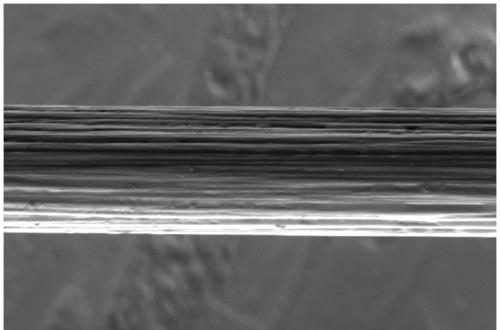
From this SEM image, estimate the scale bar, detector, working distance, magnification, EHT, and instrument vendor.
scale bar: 4000 nm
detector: ETD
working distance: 5.6 mm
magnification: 10 K X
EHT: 3 kV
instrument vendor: FEI